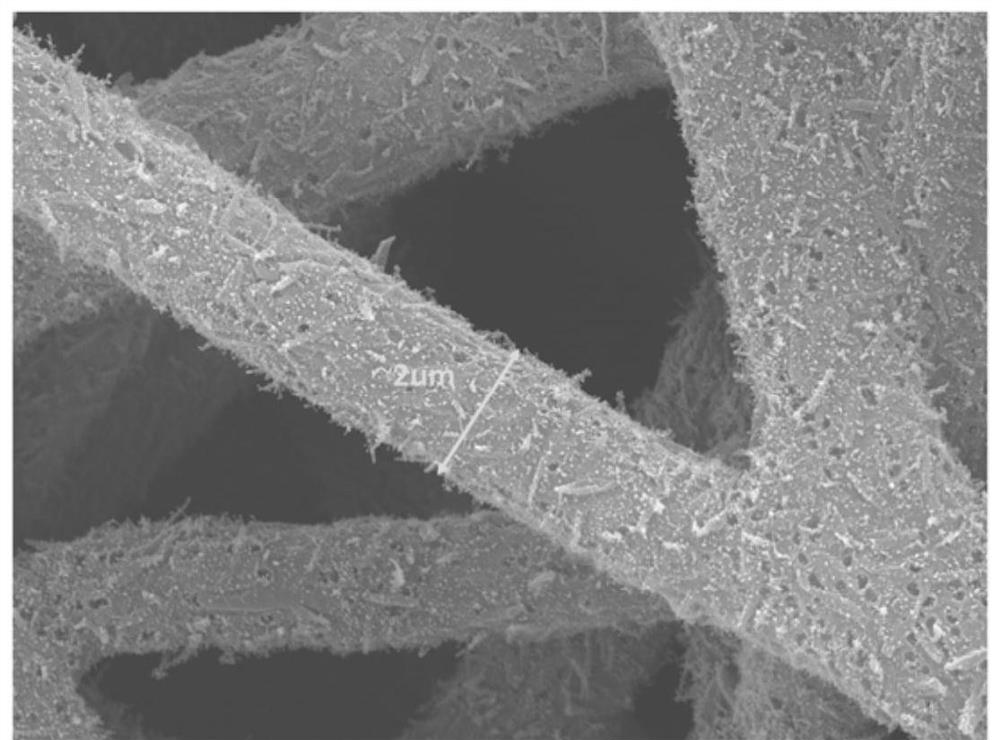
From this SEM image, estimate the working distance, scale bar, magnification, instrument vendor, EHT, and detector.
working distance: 9.8 mm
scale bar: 1000 nm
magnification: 10 K X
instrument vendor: JEOL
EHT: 10 kV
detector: LED